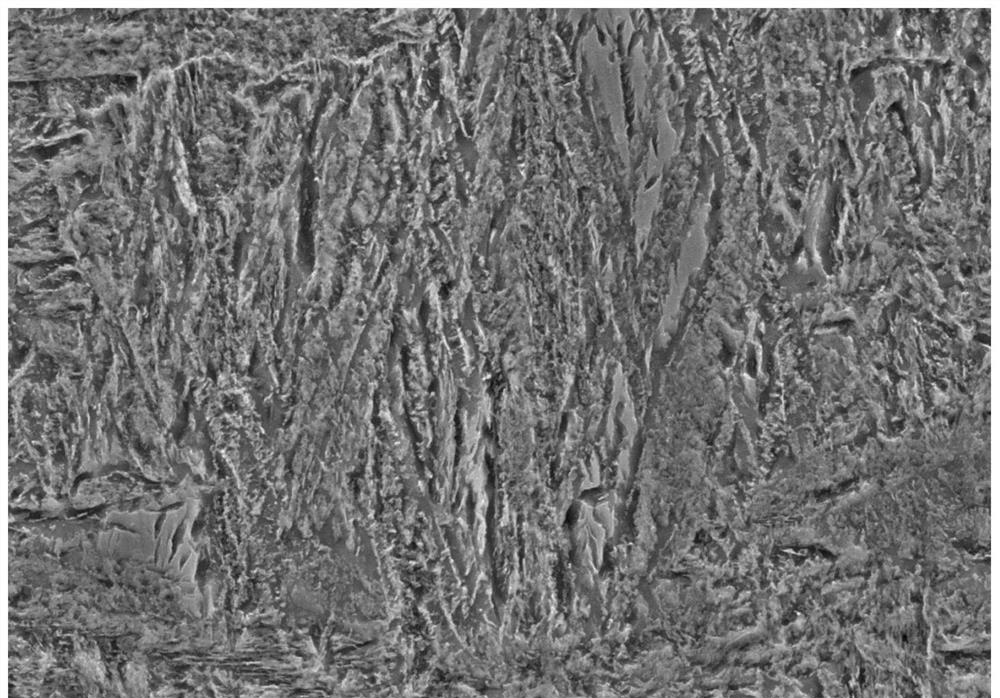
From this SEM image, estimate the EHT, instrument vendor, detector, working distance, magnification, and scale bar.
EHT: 15 kV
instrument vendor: Hitachi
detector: SE(L)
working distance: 8.4 mm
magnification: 10 K X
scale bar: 5000 nm